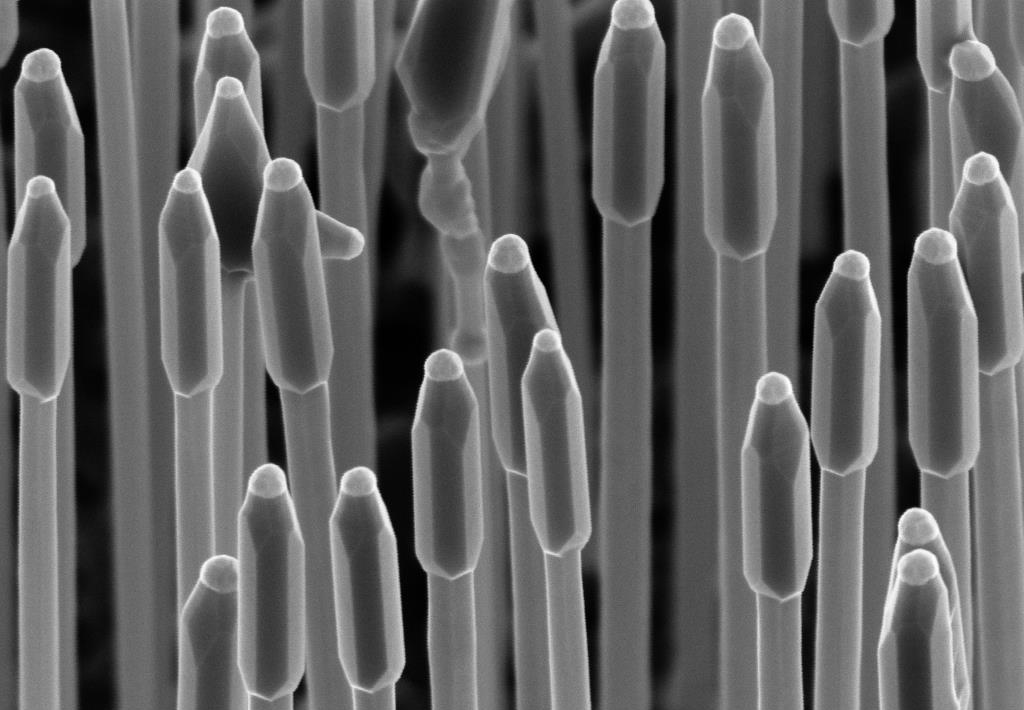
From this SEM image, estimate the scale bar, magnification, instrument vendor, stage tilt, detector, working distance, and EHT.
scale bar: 200 nm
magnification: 40 K X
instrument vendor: Zeiss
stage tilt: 45°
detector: InLens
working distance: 4.7 mm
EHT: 5 kV